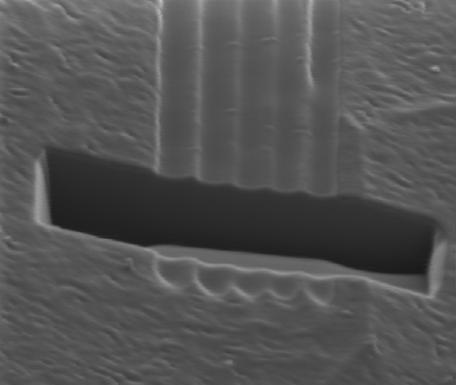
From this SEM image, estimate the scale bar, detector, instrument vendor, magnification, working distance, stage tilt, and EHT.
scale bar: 2000 nm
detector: CDM-E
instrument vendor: FEI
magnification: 25 K X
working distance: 17 mm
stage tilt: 45°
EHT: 30 kV